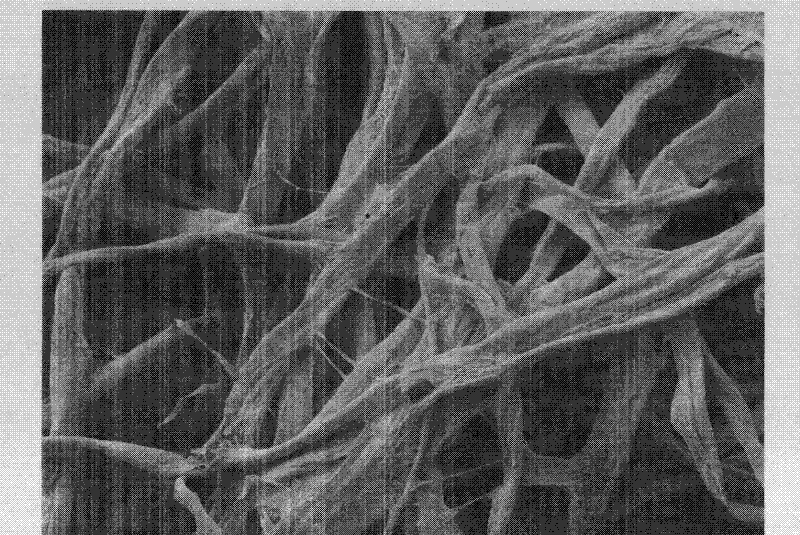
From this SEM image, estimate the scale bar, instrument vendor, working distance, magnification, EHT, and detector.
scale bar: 10000 nm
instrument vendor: JEOL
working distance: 8 mm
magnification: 0.3 K X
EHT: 5 kV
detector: LEI